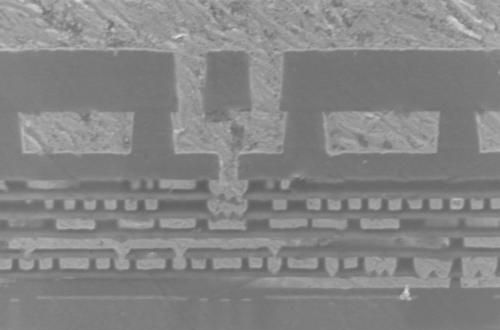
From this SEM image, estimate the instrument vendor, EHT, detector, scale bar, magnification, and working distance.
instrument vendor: Zeiss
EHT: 2 kV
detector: InLens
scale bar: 200 nm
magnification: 55 K X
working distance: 3.7 mm